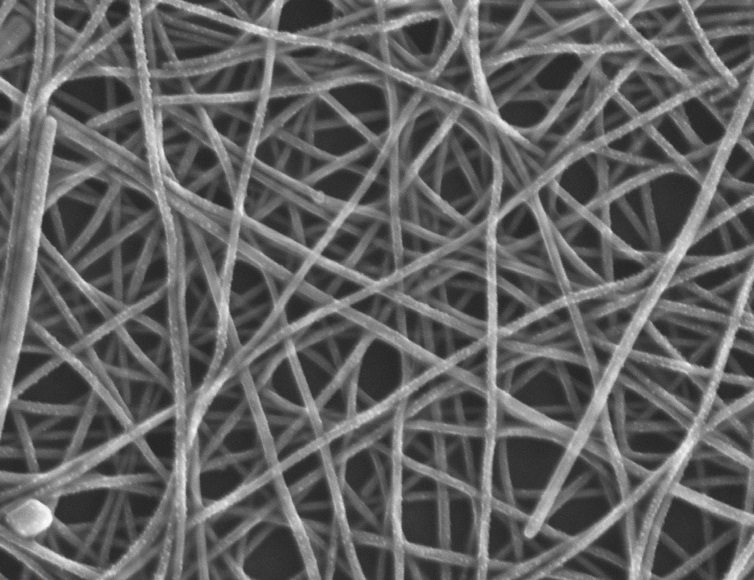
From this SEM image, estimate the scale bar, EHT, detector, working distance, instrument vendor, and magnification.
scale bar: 200 nm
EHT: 20 kV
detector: InLens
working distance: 3.1 mm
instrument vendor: Zeiss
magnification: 100 K X